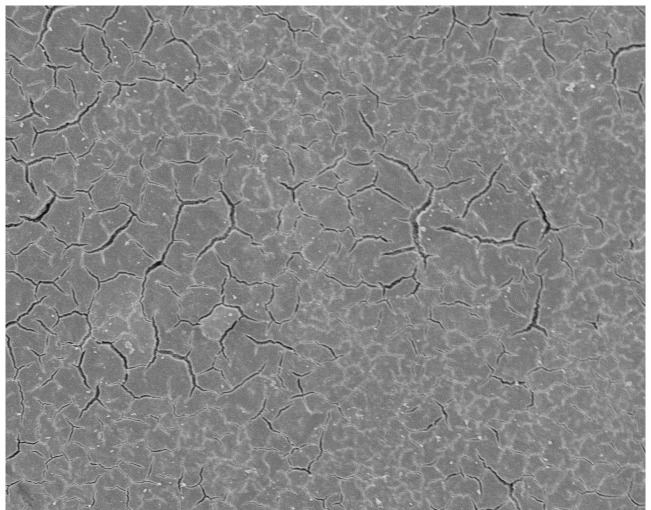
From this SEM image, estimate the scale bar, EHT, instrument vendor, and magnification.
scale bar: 100000 nm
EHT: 10 kV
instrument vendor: JEOL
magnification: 0.24 K X